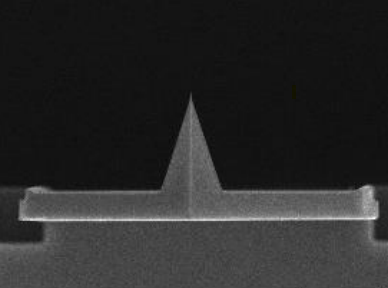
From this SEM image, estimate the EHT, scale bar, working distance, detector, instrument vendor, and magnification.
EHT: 20 kV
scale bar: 10000 nm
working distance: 14.5 mm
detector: SEI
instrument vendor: JEOL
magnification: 2.5 K X